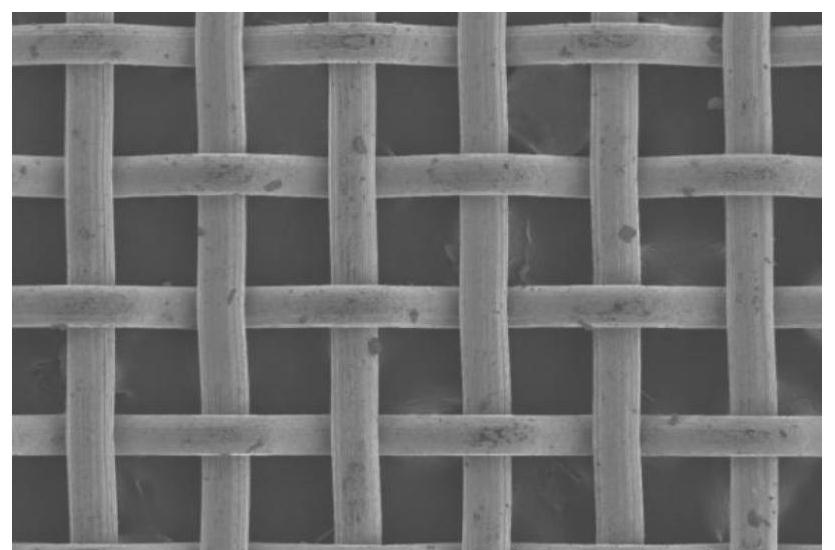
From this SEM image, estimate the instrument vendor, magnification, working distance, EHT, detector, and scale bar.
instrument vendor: Hitachi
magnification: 0.1 K X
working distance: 9.8 mm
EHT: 3 kV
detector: SE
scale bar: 500000 nm